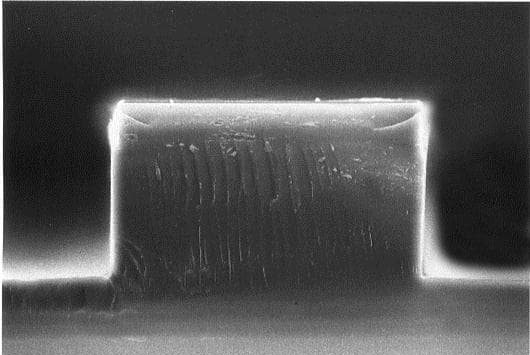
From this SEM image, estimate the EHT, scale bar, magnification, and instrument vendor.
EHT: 15 kV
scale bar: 10000 nm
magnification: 1.5 K X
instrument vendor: JEOL